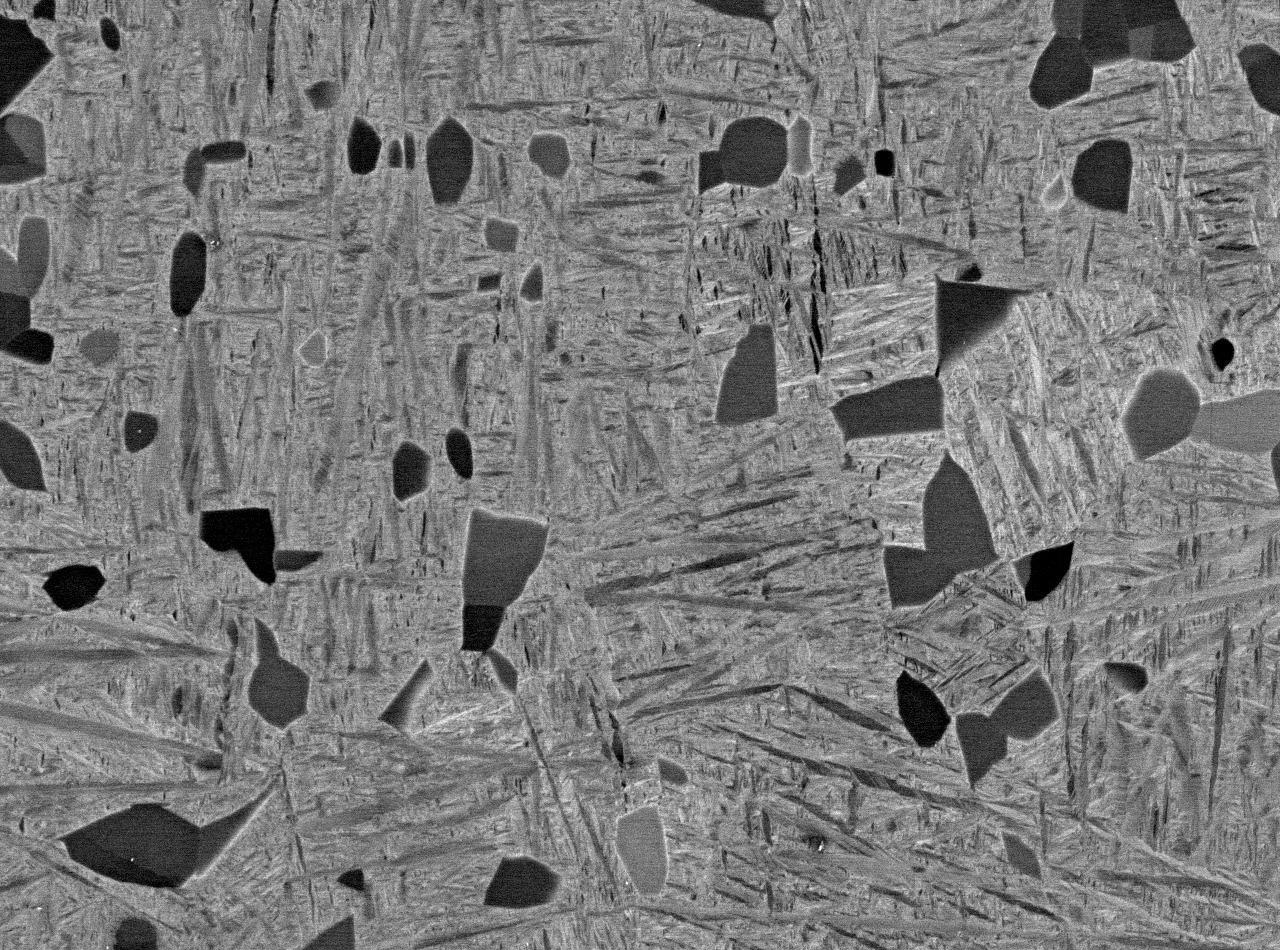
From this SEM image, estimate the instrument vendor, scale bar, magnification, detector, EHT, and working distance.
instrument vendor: JEOL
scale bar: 10000 nm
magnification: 1.5 K X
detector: COMPO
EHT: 15 kV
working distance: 10 mm